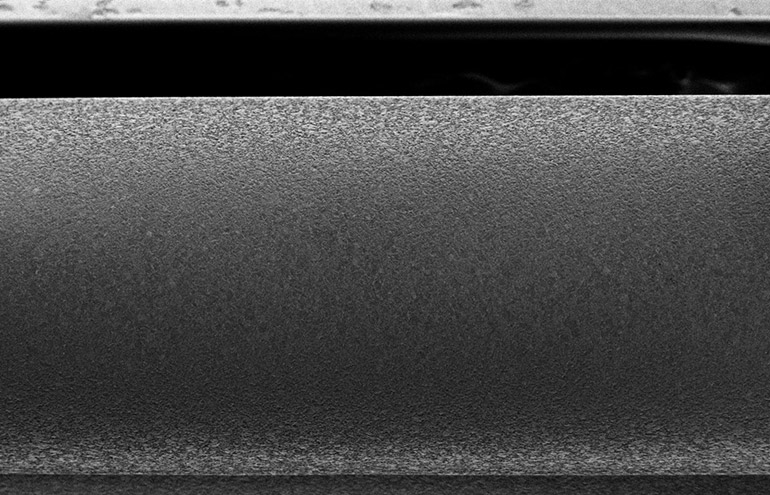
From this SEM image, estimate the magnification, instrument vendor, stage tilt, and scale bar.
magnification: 0.2 K X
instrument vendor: other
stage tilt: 0°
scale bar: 100000 nm